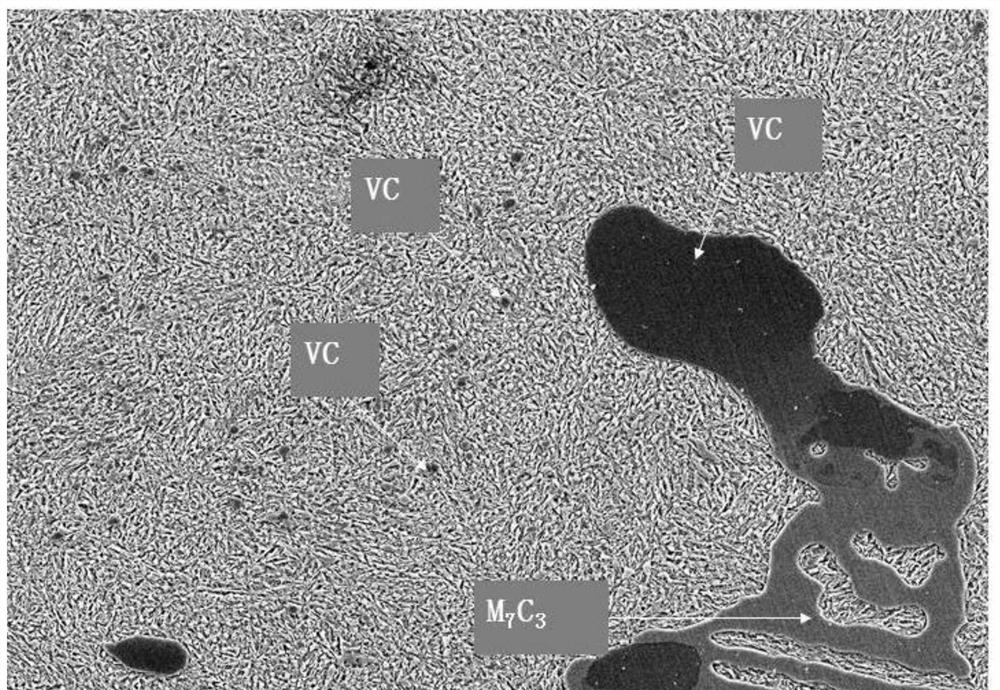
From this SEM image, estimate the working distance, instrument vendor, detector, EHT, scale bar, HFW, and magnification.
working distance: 11.2 mm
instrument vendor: JEOL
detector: SED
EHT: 10 kV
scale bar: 5000 nm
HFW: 64 µm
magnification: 2 K X